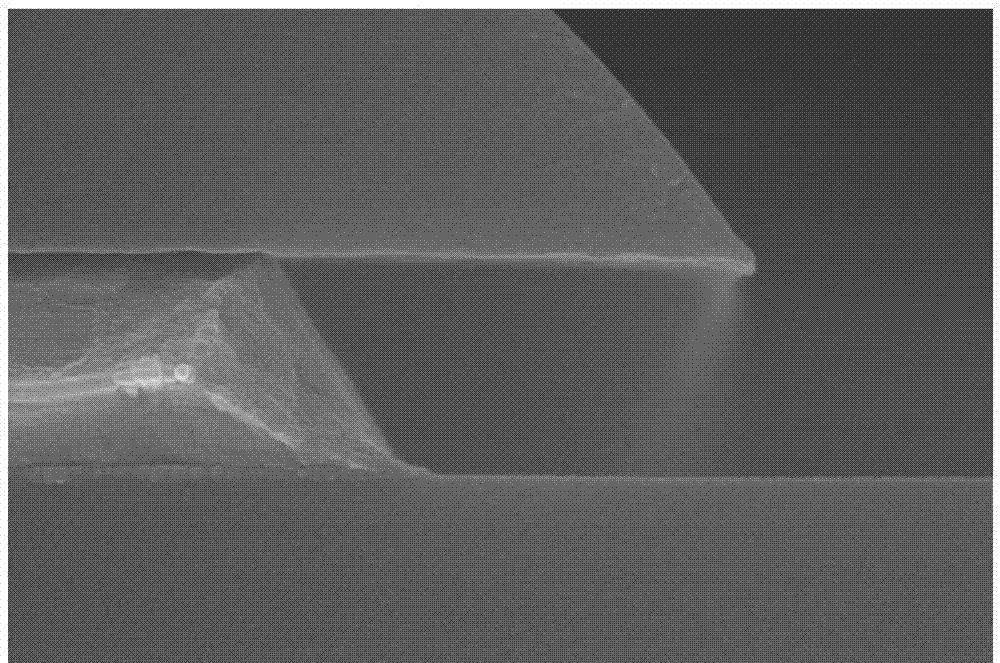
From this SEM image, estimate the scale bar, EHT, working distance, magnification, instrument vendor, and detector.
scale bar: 500 nm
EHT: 15 kV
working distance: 8 mm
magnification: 60 K X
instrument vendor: Hitachi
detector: SE(U,LA0)